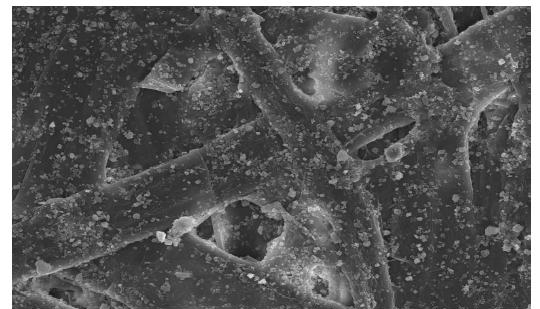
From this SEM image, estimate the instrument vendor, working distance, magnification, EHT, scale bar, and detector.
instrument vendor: Hitachi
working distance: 12.1 mm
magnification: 1 K X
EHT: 15 kV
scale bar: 50000 nm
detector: SE(U)